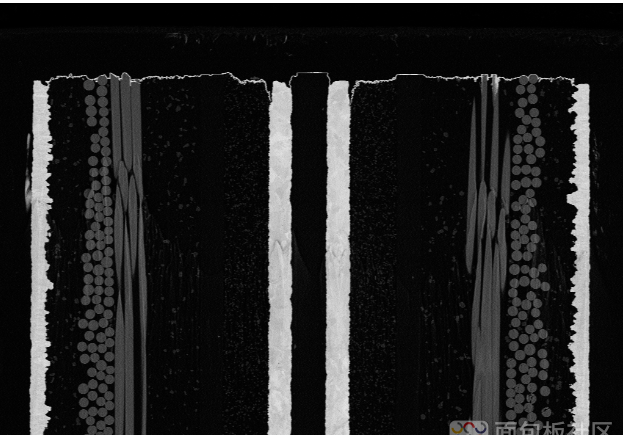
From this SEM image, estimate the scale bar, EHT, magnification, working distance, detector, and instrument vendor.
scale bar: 10000 nm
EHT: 8 kV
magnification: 0.35 K X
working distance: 5.6 mm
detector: NTS BSD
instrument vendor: Zeiss